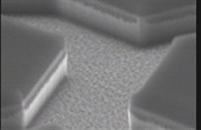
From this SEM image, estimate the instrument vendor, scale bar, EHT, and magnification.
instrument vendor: JEOL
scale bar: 2000 nm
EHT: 15 kV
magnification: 9.5 K X